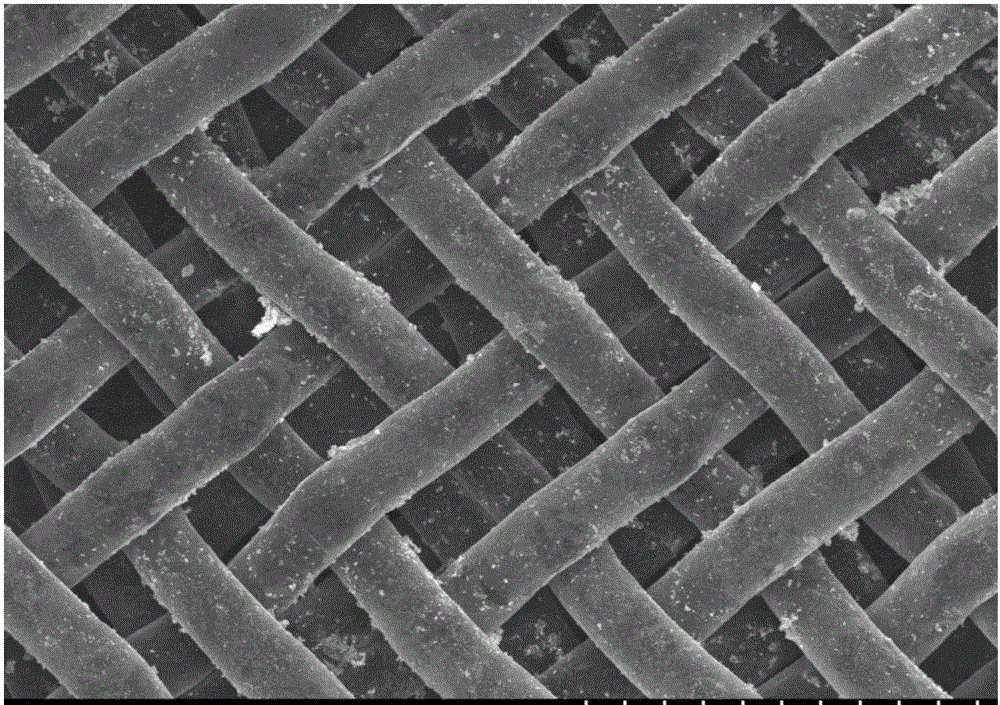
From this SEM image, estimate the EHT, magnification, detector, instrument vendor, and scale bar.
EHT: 8 kV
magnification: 0.25 K X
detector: SE(M)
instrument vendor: Hitachi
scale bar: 200000 nm